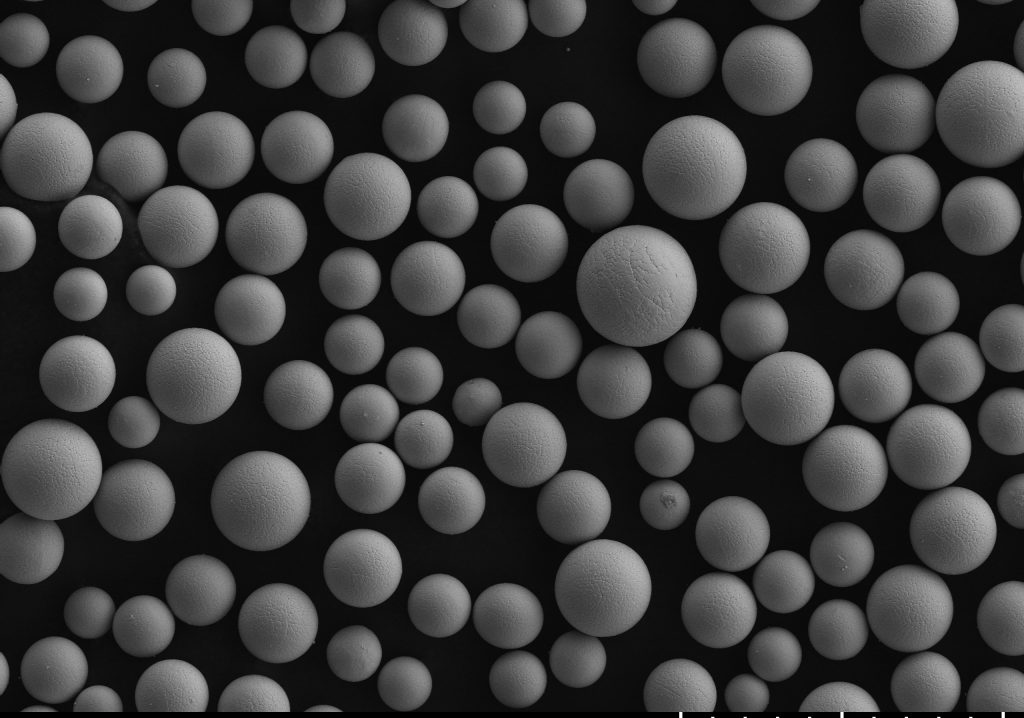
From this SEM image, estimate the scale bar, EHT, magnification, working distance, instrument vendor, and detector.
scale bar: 200000 nm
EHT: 15 kV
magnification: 0.2 K X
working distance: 15 mm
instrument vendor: Hitachi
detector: SE(L)